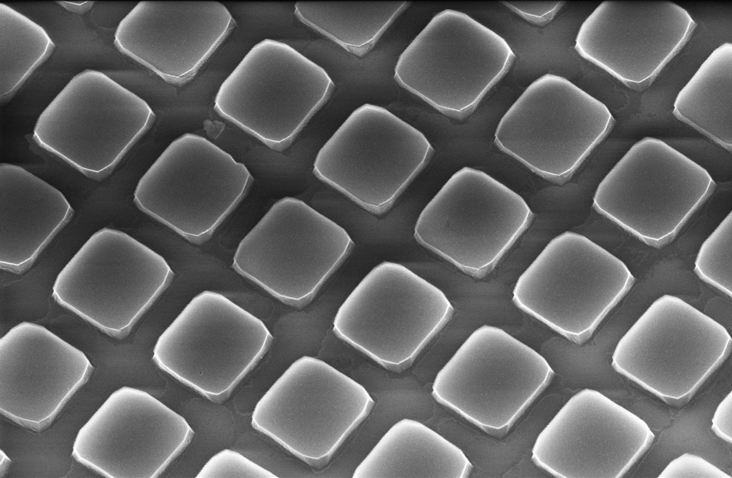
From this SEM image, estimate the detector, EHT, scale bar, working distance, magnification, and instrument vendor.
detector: InLens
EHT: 10 kV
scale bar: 1000 nm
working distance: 8.1 mm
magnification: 12 K X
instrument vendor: Zeiss